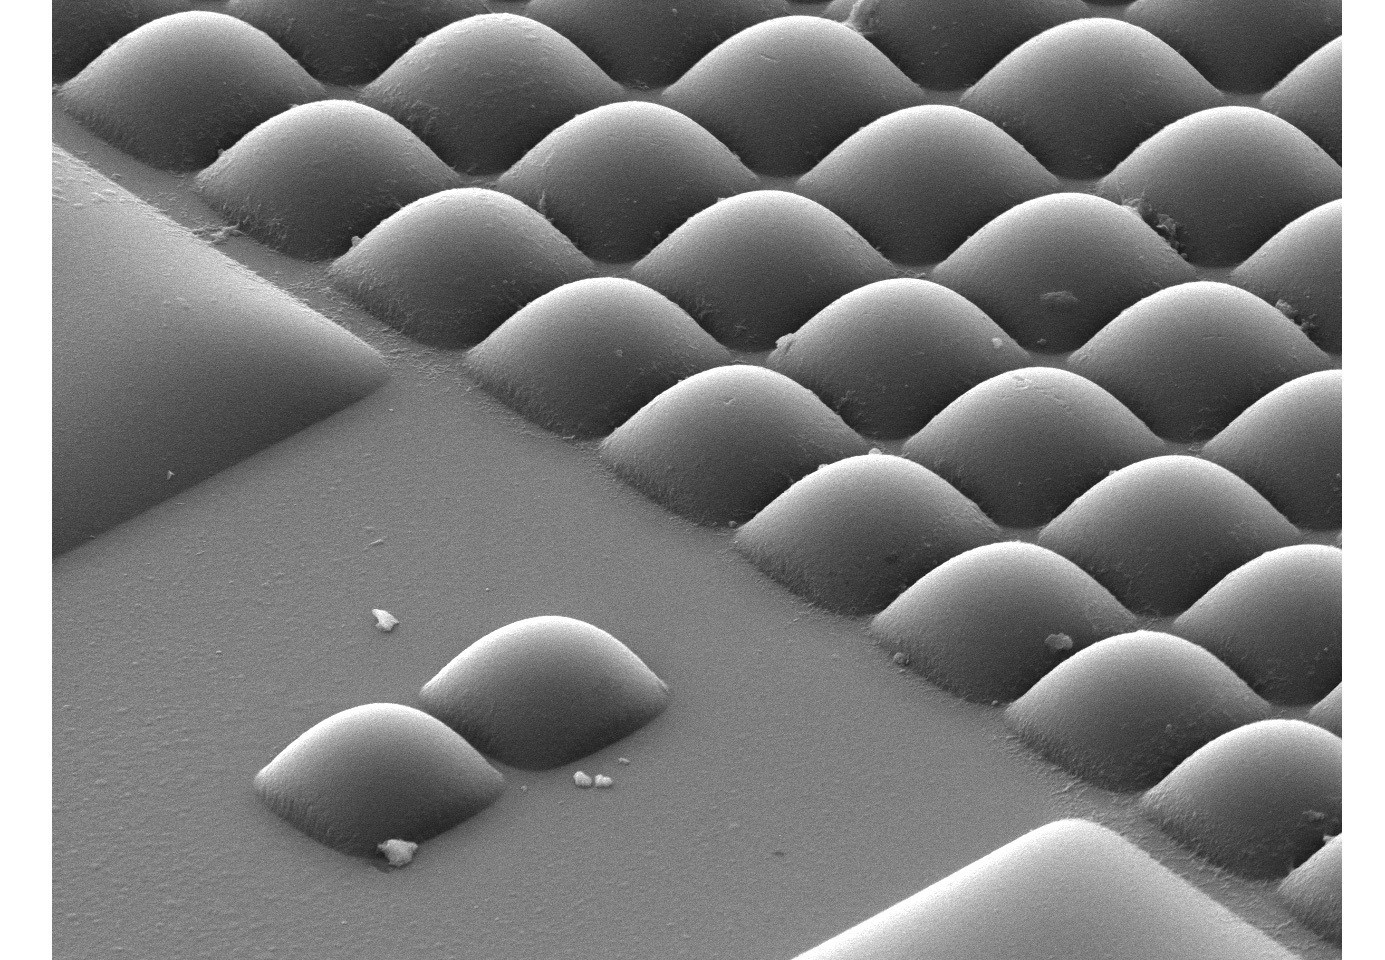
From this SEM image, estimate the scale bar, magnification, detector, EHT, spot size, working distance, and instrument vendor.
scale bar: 5000 nm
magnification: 3.5 K X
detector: SE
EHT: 10 kV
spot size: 3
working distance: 6.4 mm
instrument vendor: FEI